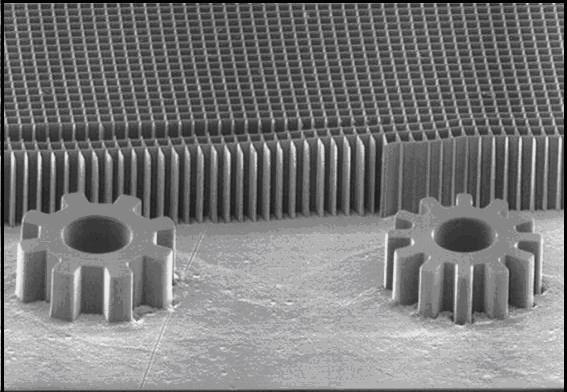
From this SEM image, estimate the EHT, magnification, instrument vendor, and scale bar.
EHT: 20 kV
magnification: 0.1 K X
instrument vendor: other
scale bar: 100000 nm